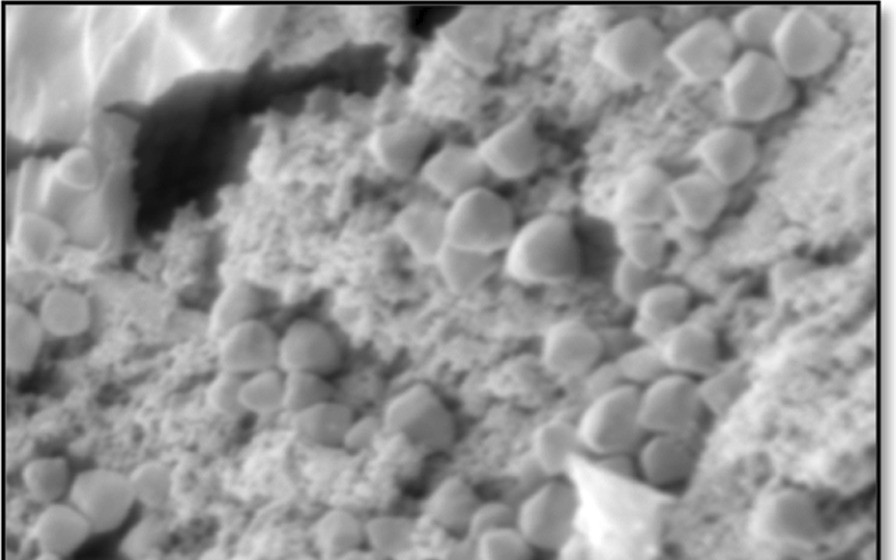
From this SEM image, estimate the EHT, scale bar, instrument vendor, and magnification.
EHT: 10 kV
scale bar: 5000 nm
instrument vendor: JEOL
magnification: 3.5 K X